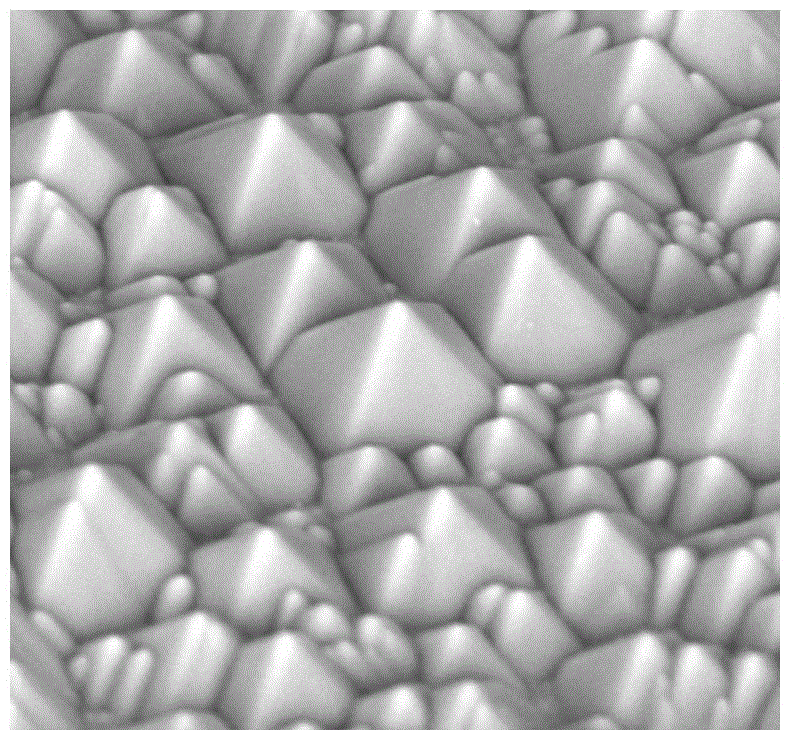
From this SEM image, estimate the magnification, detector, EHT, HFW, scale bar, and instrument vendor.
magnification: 11 K X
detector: BSD Full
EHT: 10 kV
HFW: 24.4 µm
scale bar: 5000 nm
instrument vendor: Thermo